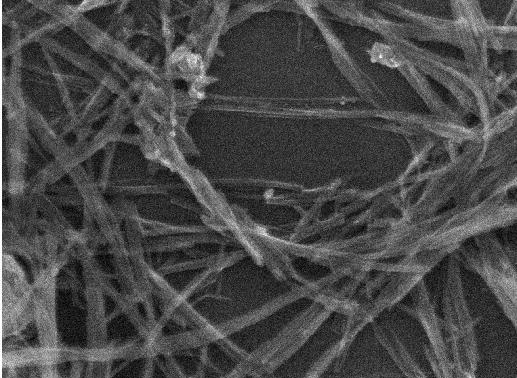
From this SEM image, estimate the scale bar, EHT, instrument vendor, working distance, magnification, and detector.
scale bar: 100 nm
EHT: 10 kV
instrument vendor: JEOL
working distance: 7.5 mm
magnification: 100 K X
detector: SEI